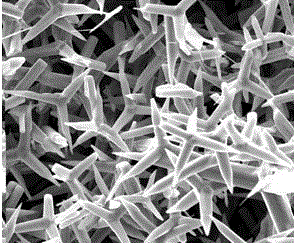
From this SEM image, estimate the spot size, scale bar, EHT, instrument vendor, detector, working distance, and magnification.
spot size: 3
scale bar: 20000 nm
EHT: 20 kV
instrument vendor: FEI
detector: ETD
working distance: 9.1 mm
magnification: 5 K X